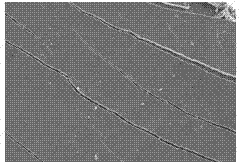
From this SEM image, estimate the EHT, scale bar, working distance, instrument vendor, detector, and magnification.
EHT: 15 kV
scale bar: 100000 nm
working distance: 10.2 mm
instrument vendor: Hitachi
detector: SE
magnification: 0.5 K X